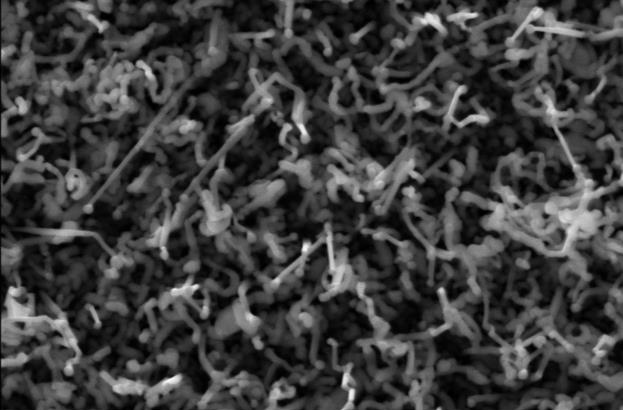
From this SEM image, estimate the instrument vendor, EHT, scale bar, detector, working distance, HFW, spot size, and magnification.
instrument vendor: FEI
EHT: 20 kV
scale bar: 3000 nm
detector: ETD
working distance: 11.7 mm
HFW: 8.29 µm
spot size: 5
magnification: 50 K X